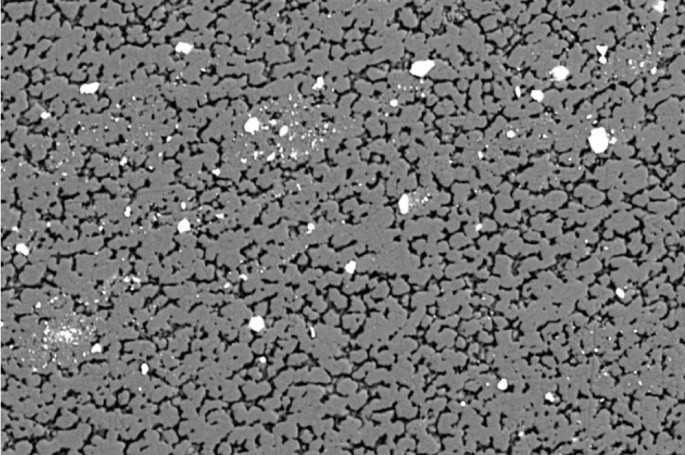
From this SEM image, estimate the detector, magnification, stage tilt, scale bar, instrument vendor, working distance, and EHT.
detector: NTS BSD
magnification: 2 K X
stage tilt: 0°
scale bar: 10000 nm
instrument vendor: Zeiss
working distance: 8.5 mm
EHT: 20 kV